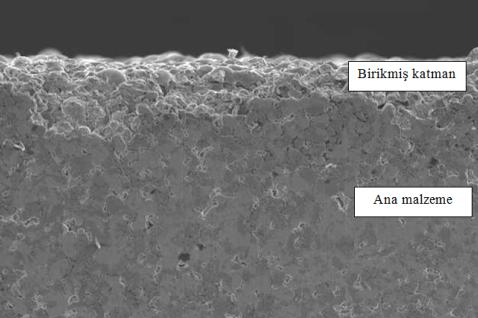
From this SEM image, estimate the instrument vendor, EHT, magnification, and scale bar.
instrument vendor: JEOL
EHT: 20 kV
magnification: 0.1 K X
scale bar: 100000 nm